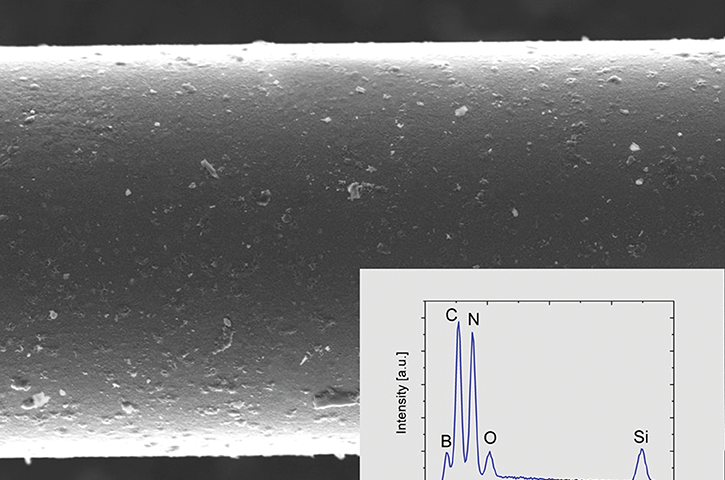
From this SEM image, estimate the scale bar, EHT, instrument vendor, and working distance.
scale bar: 2000 nm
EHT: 3 kV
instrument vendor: Zeiss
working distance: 4.5 mm